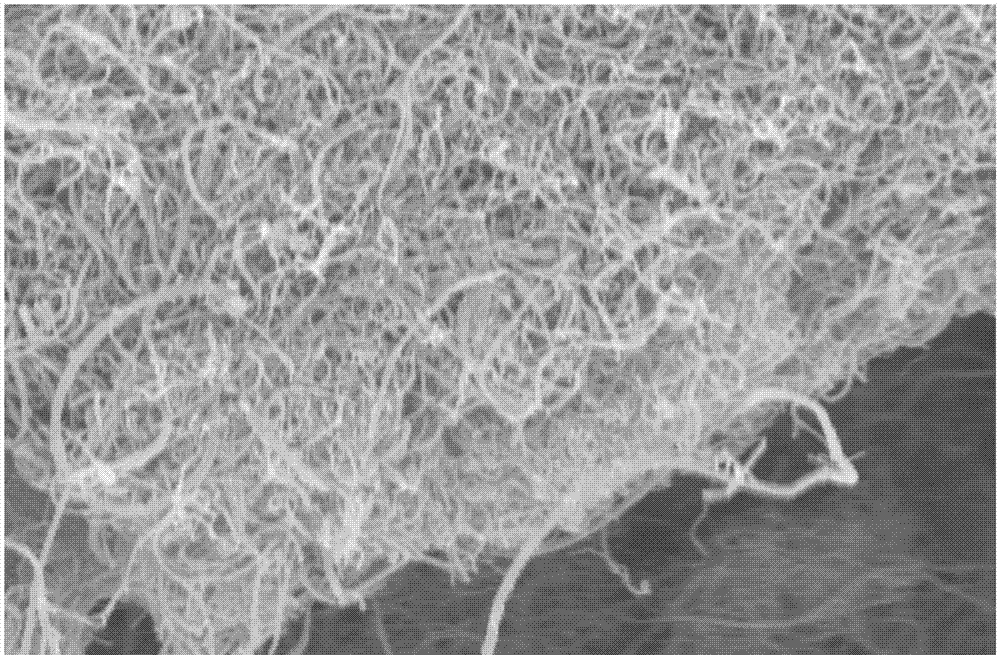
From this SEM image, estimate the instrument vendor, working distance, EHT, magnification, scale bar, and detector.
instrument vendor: Zeiss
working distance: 5.7 mm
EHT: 5 kV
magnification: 16.16 K X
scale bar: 1000 nm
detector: InLens